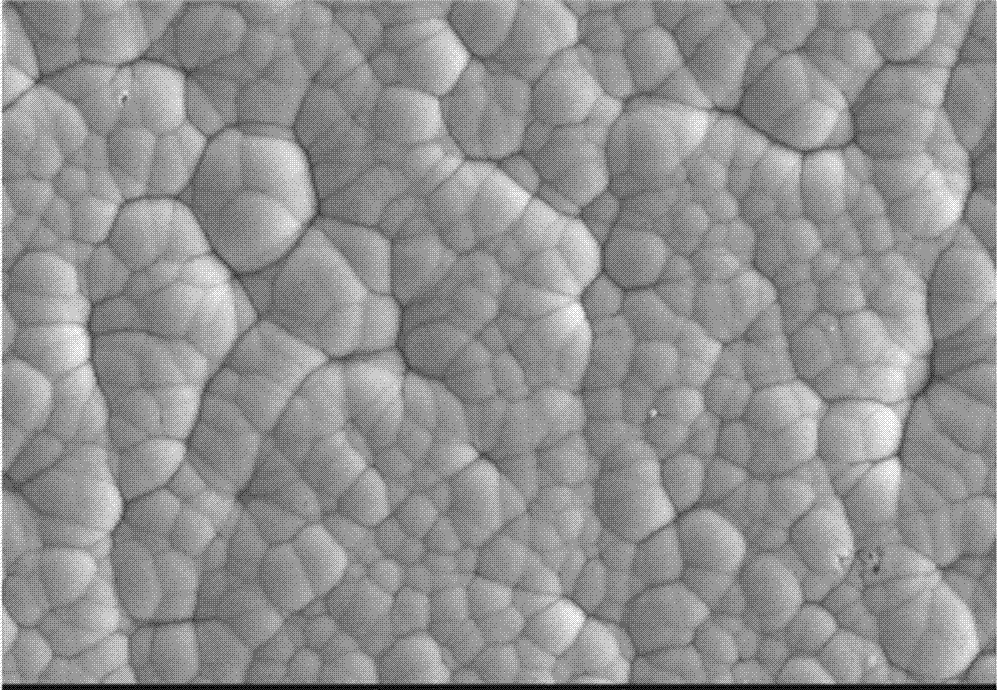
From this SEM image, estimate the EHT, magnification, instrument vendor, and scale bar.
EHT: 15 kV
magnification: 3 K X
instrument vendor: other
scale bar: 10000 nm